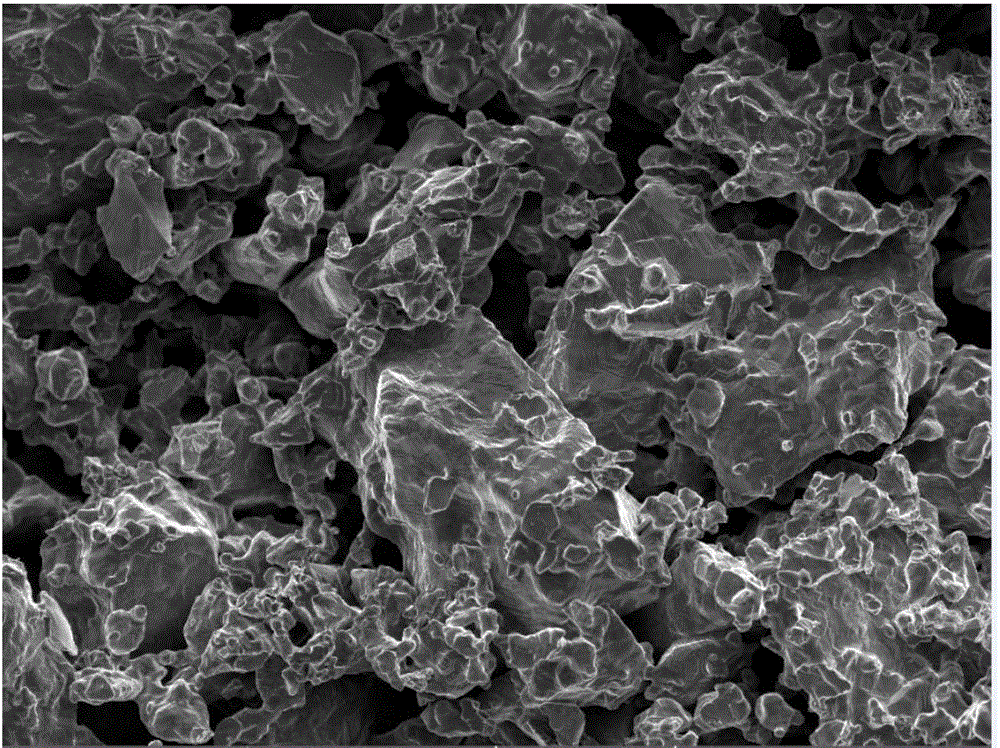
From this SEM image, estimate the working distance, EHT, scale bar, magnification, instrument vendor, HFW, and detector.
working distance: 4.98 mm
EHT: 10 kV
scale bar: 50000 nm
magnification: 1 K X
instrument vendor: Tescan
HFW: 277 µm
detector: InBeam SE